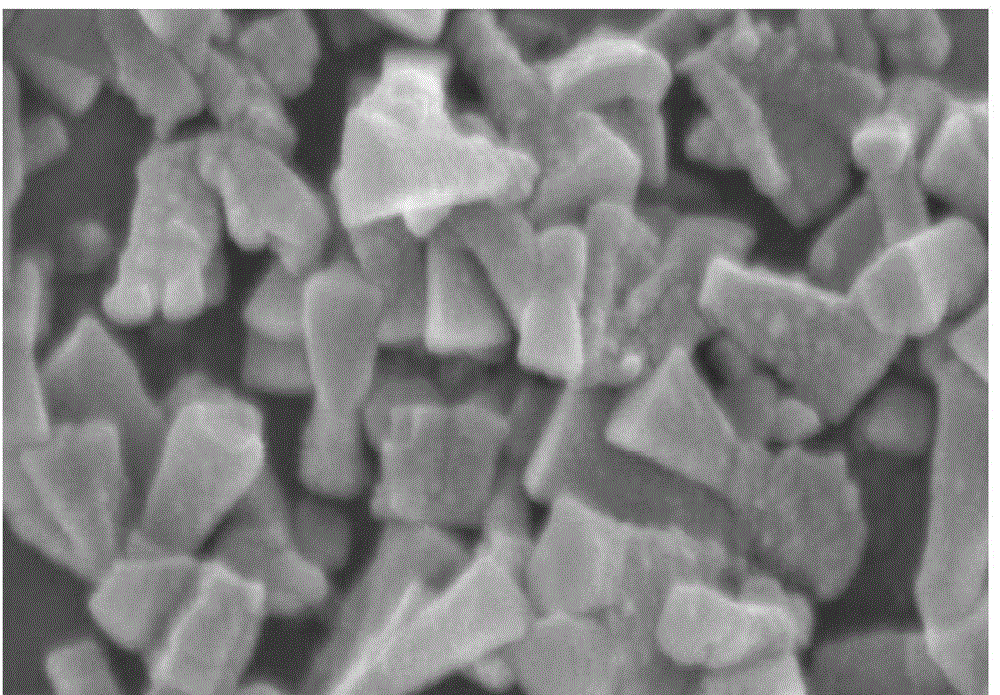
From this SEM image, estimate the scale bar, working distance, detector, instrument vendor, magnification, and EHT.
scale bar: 500 nm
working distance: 7 mm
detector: SE(U)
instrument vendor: Hitachi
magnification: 100 K X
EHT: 3 kV